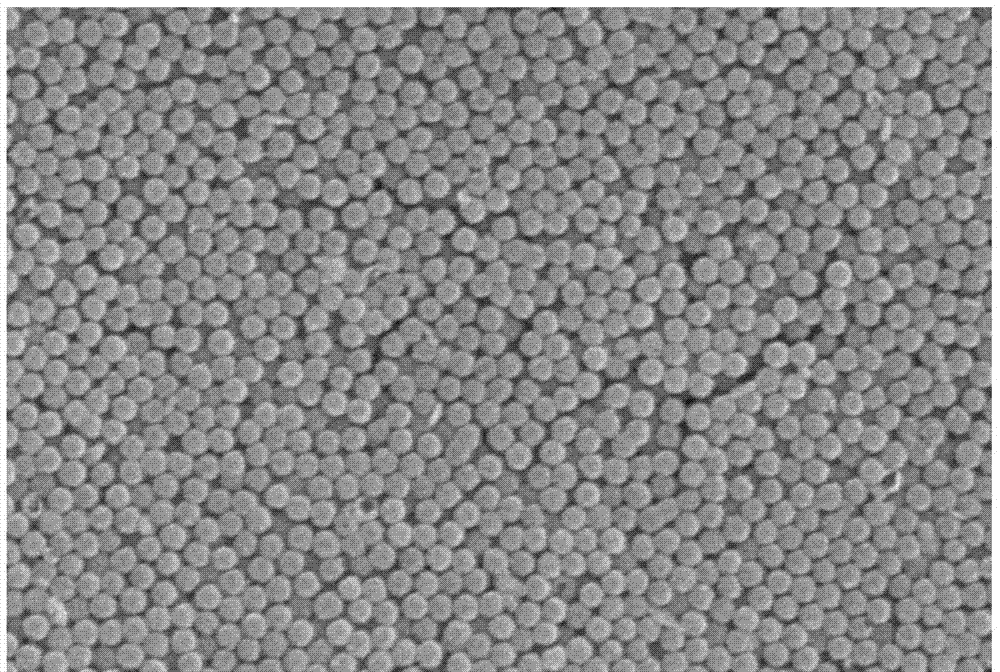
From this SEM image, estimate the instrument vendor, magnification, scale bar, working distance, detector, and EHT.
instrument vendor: Hitachi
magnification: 20 K X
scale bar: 2000 nm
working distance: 15.8 mm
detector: SE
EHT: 10 kV